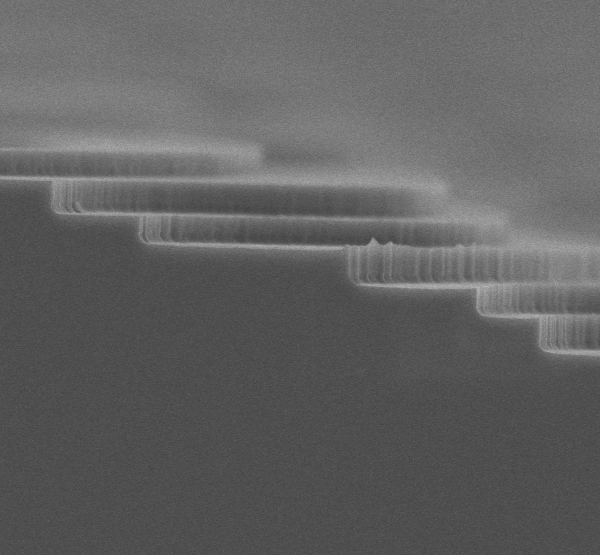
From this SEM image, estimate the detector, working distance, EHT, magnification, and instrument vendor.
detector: SE(UL)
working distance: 10 mm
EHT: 3 kV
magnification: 20 K X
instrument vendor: Hitachi